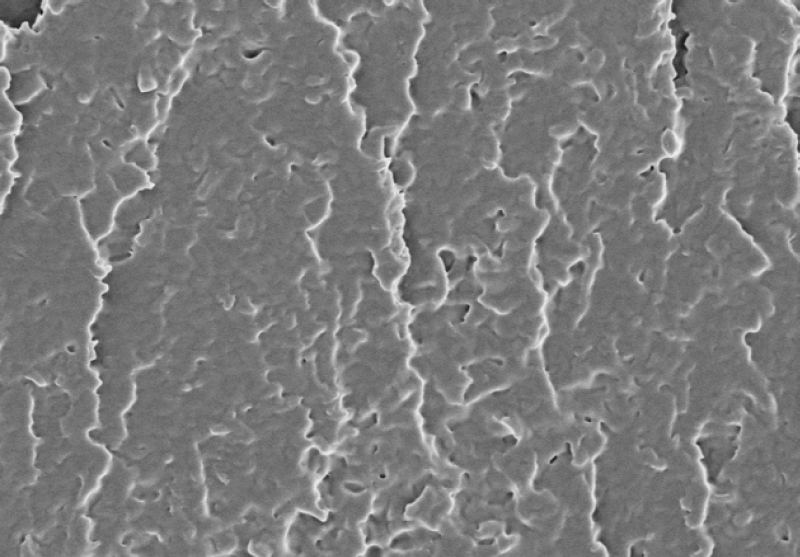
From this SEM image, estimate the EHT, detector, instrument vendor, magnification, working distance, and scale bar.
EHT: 3 kV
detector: SE(M)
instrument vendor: Hitachi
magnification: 5 K X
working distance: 10 mm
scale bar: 10000 nm